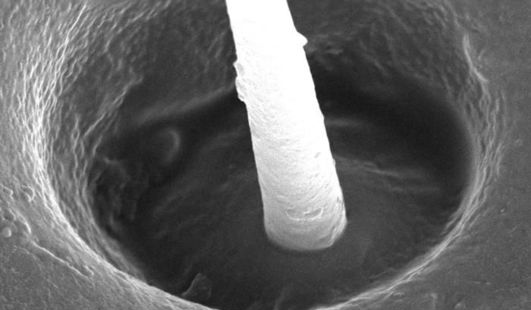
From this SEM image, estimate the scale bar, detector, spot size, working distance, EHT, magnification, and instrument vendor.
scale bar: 5000 nm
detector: SE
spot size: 3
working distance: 6.6 mm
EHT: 30 kV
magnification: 3.862 K X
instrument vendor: FEI